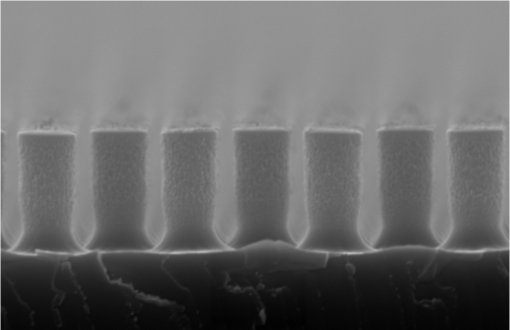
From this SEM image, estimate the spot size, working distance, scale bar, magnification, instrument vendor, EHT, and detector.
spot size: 50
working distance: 10 mm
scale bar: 2000 nm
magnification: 9 K X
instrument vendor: JEOL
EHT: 20 kV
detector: SEI